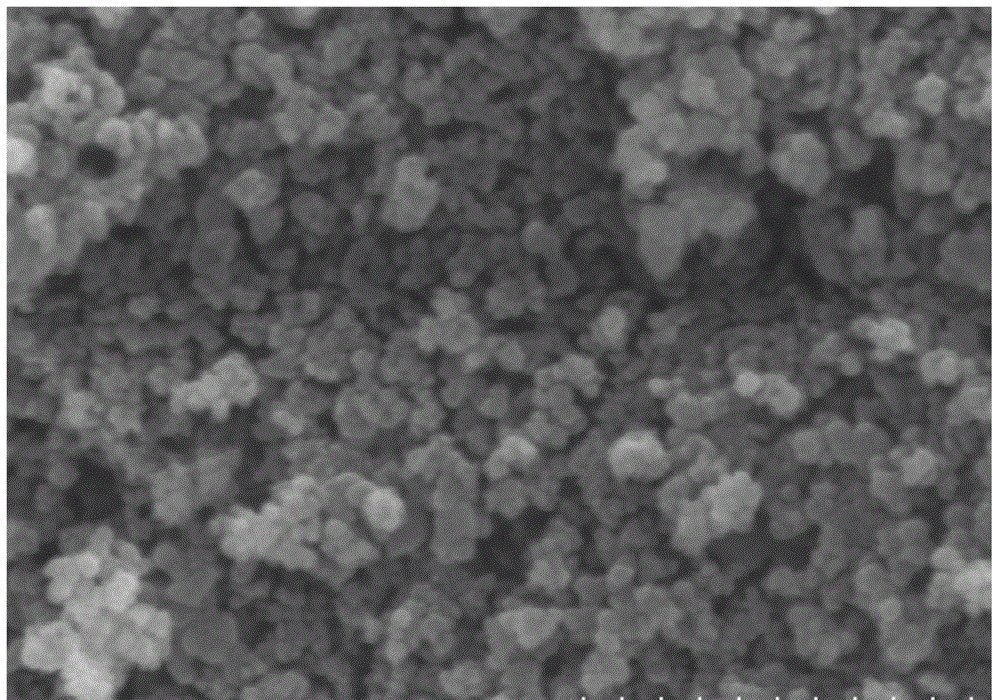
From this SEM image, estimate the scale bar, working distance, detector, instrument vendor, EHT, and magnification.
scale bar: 500 nm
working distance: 8 mm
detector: SE(M)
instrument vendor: Hitachi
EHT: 10 kV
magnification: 100 K X